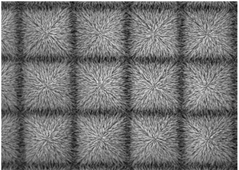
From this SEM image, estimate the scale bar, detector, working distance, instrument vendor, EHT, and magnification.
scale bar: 1000 nm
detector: SEI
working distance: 4.4 mm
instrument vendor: JEOL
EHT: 5 kV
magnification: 3.5 K X